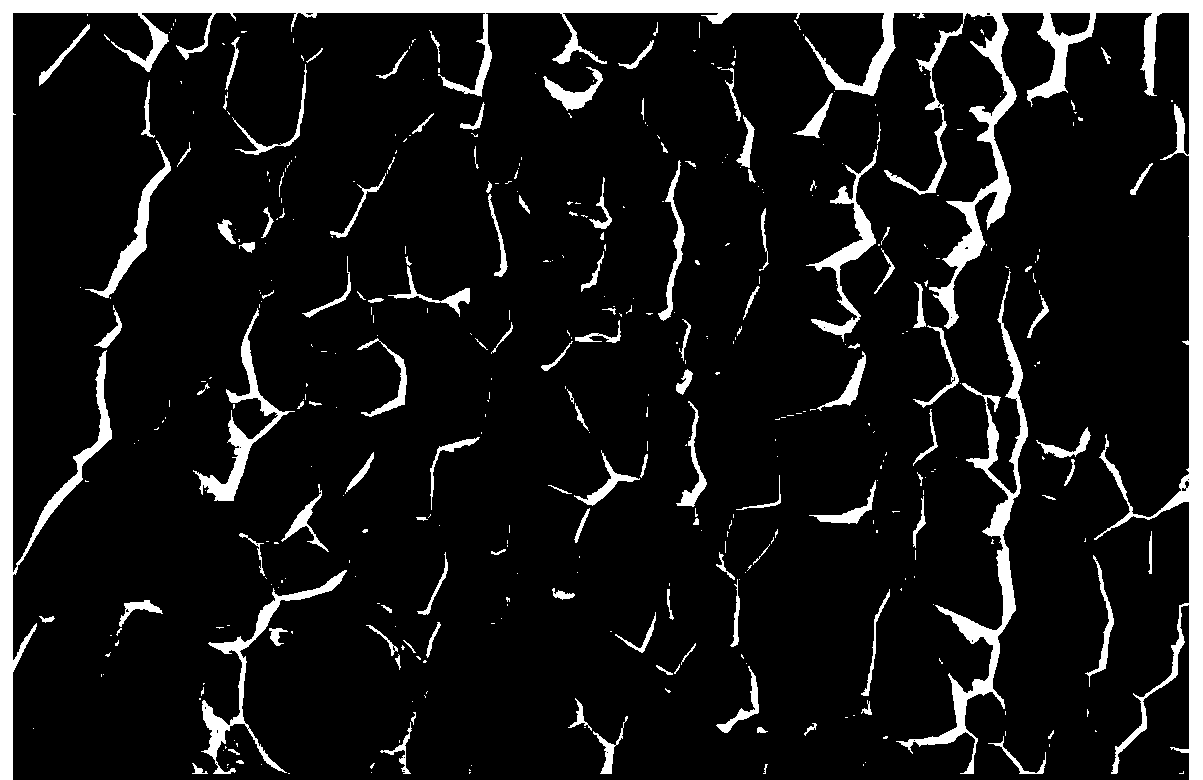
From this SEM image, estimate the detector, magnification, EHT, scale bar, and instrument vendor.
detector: SEI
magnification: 0.1 K X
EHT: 15 kV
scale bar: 100000 nm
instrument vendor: JEOL